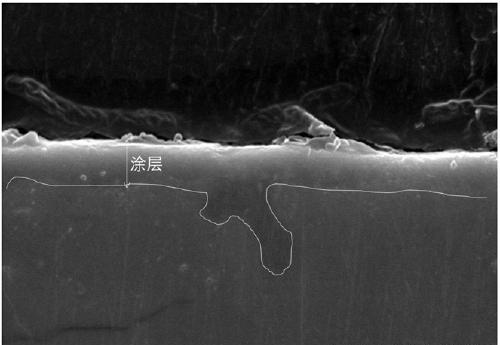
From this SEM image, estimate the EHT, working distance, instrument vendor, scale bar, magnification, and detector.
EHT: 20 kV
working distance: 14.9 mm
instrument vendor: Hitachi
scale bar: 5000 nm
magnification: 8 K X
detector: SE(U,LA0)